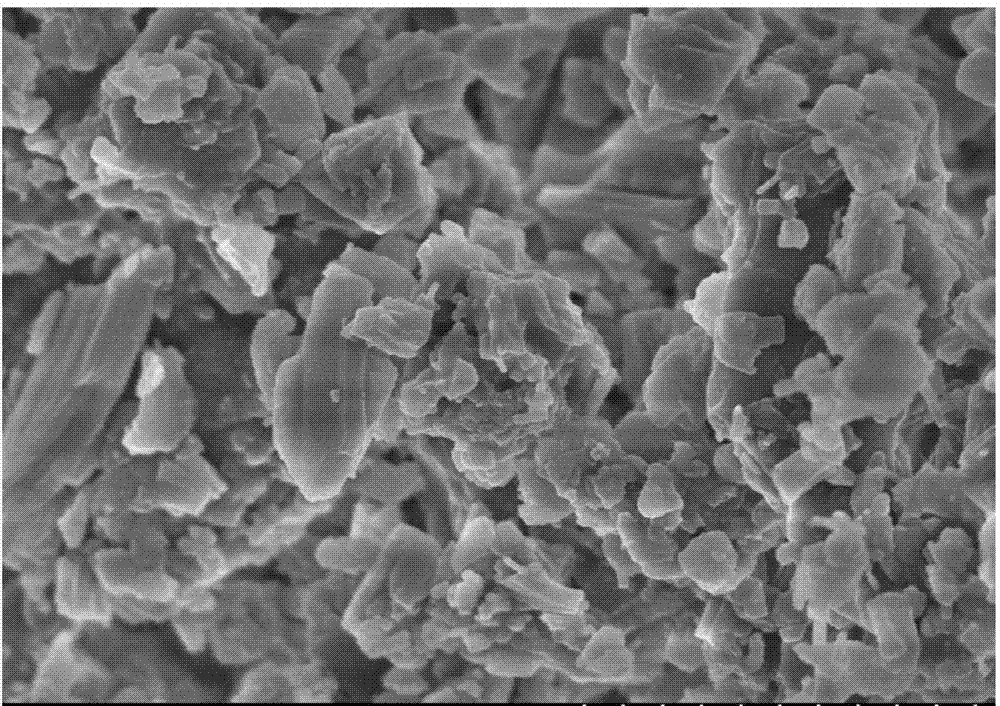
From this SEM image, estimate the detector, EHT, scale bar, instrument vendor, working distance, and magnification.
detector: SE(U,LA0)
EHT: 3 kV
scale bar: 2000 nm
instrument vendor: Hitachi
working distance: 3.9 mm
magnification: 25 K X